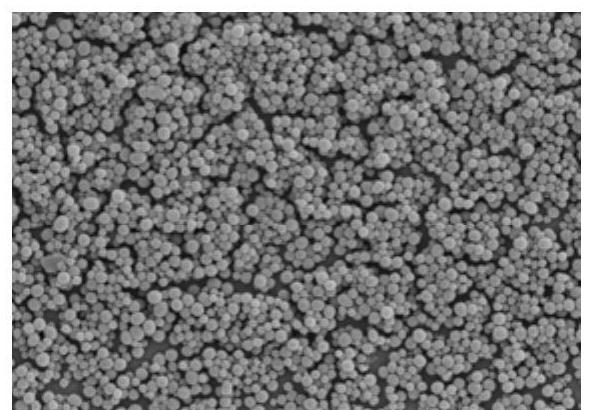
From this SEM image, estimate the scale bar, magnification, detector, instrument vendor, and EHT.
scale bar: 2000 nm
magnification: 20 K X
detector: SE(UL)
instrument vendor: Hitachi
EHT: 4 kV